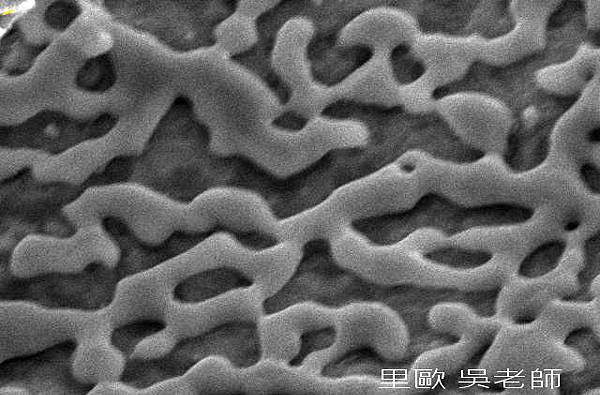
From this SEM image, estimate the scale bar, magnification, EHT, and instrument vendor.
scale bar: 2000 nm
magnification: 6 K X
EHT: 20 kV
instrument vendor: JEOL